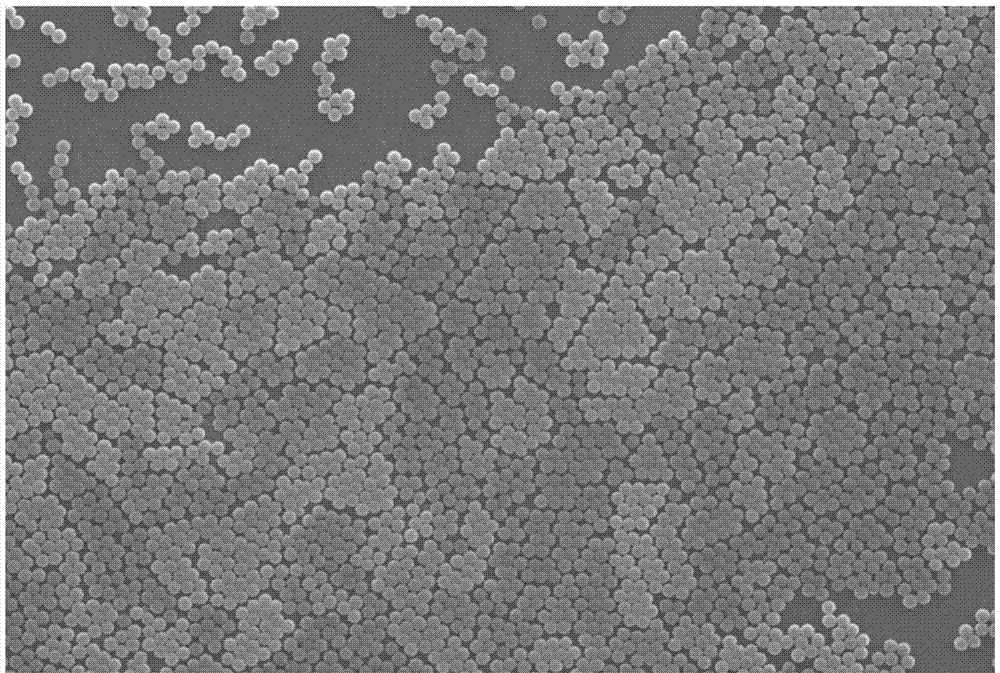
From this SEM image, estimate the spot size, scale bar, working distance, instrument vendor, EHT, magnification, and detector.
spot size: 32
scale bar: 5000 nm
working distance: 10 mm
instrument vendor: JEOL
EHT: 20 kV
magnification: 5 K X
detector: SEI